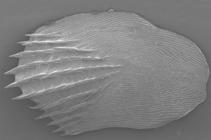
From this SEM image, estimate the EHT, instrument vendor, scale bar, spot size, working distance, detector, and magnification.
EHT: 12.5 kV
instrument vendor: FEI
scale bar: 2000000 nm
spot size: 4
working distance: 19.5 mm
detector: SE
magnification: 0.057 K X